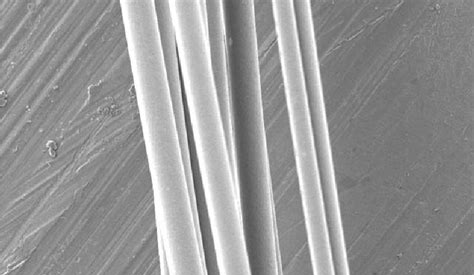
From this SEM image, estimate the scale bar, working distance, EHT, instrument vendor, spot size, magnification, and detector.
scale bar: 50000 nm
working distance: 8.8 mm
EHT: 5 kV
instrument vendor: FEI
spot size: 5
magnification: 0.5 K X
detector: SE